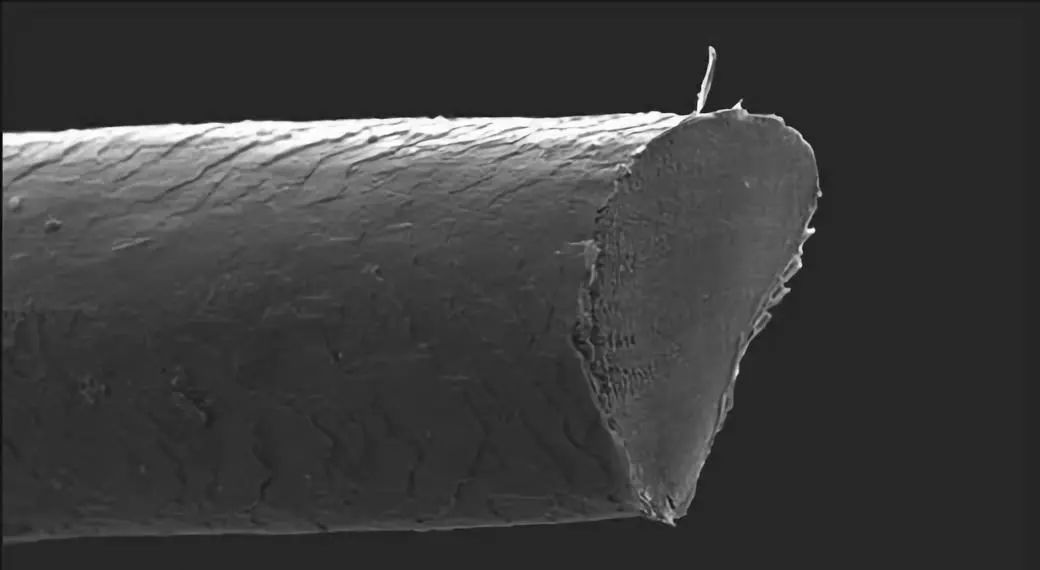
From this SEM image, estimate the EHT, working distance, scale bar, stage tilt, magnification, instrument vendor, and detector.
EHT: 5 kV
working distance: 12.6 mm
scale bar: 10000 nm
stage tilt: -0.1°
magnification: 2.7 K X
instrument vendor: Zeiss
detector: SE2